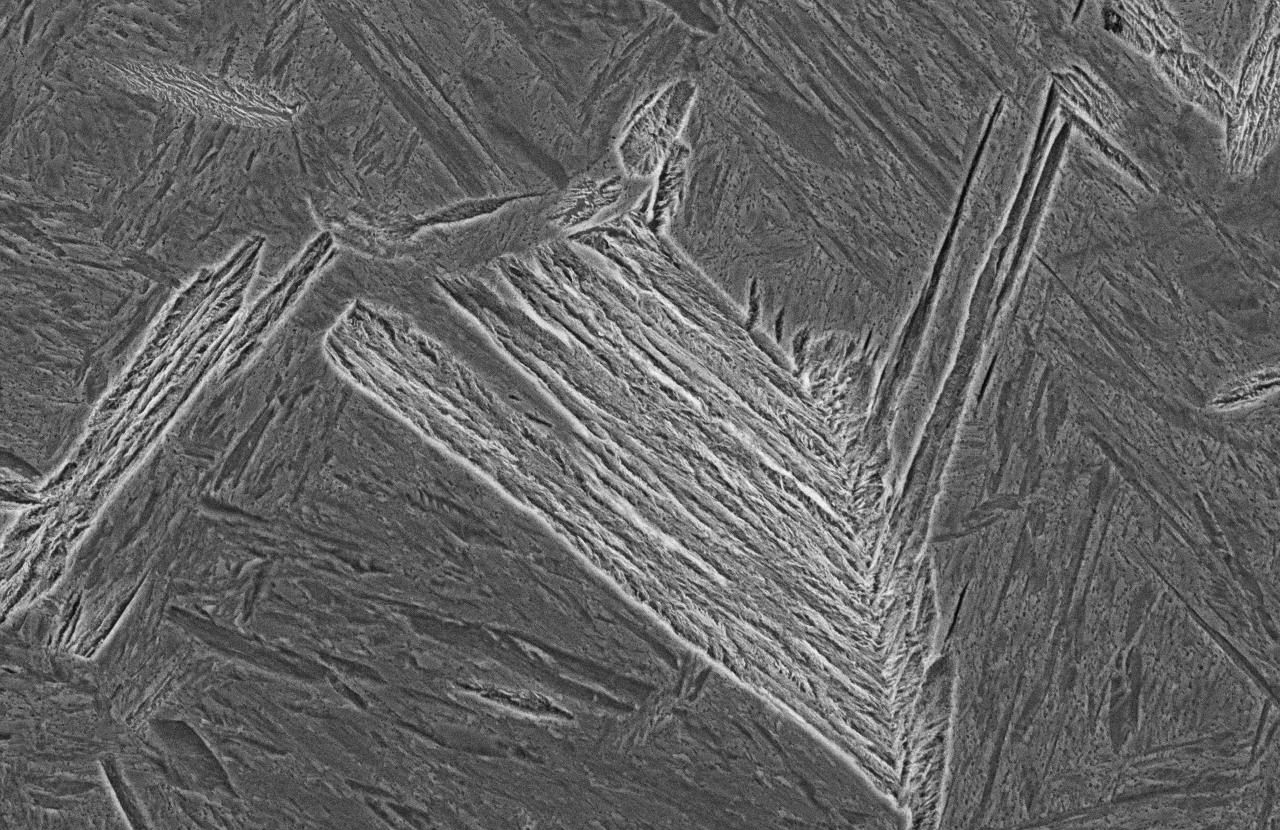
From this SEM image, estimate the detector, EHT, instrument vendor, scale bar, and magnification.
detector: SEI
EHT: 15 kV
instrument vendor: JEOL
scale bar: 20000 nm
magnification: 1.5 K X